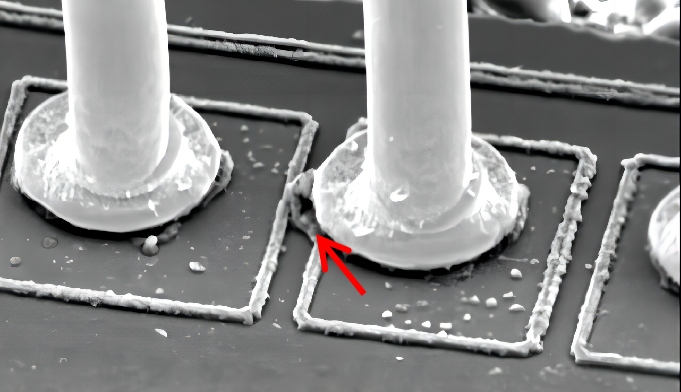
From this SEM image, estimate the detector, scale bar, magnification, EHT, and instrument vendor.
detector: SEI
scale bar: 20000 nm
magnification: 0.95 K X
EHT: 15 kV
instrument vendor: JEOL